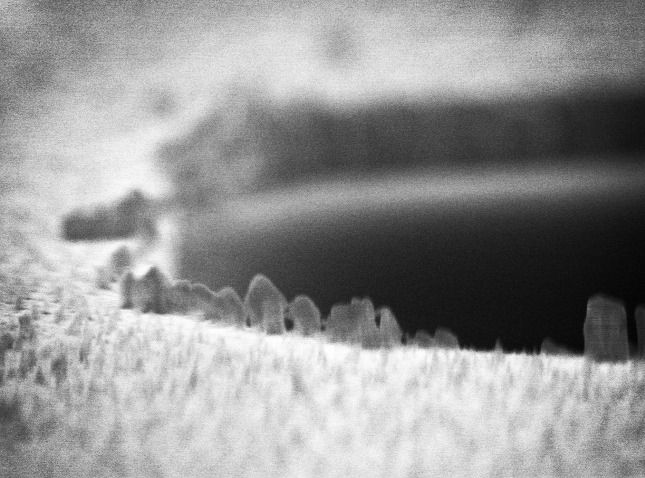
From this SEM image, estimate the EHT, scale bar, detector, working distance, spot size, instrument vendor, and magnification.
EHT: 10 kV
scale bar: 500 nm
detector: TLD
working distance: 4.3 mm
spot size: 2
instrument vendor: FEI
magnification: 41.8 K X